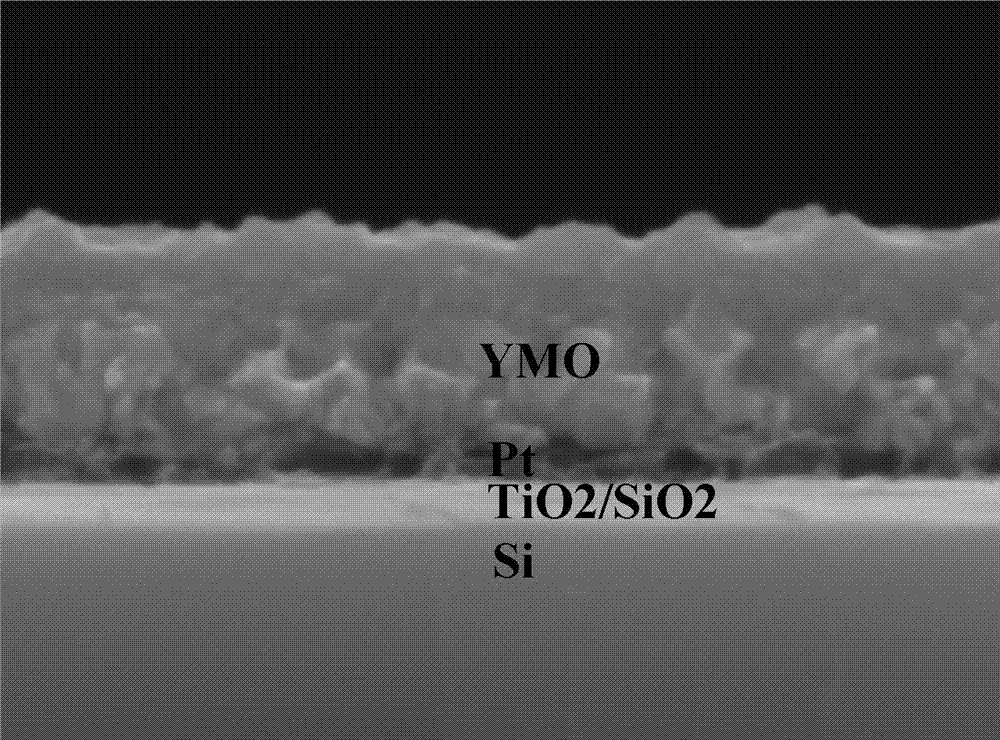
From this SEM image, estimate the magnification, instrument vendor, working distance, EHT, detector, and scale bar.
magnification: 100 K X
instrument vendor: JEOL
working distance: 9.7 mm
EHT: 10 kV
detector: SEI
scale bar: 100 nm